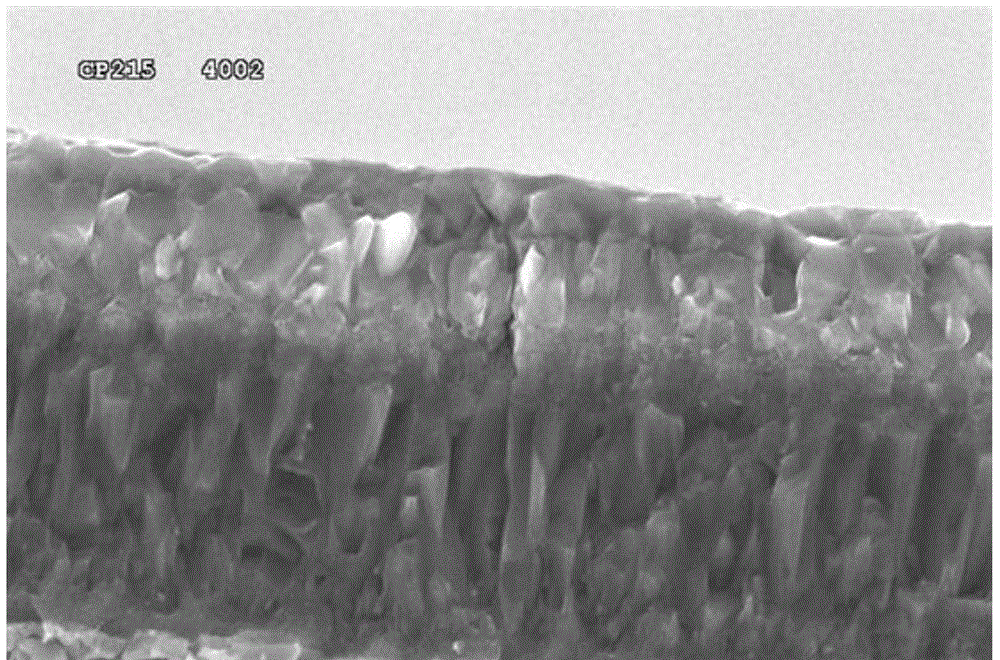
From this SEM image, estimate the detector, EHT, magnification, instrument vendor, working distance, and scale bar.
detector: SE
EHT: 20 kV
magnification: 3.5 K X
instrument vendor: Hitachi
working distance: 14.9 mm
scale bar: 10000 nm